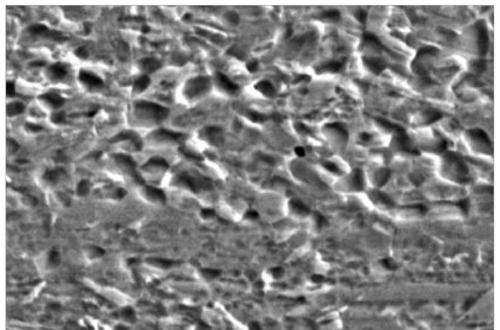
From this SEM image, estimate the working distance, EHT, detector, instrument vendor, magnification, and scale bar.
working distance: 12.7 mm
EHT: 15 kV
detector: SE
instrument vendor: JEOL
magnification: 5 K X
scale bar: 8000 nm